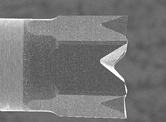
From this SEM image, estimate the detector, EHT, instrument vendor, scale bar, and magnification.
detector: SEI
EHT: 10 kV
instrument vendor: JEOL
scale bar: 100000 nm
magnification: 0.15 K X